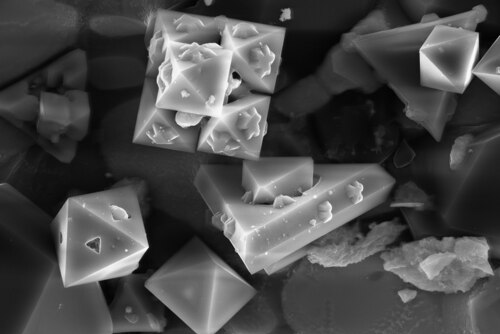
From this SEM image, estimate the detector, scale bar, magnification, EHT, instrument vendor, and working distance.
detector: InLens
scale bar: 10000 nm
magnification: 5.29 K X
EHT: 20 kV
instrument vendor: Zeiss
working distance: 6.7 mm